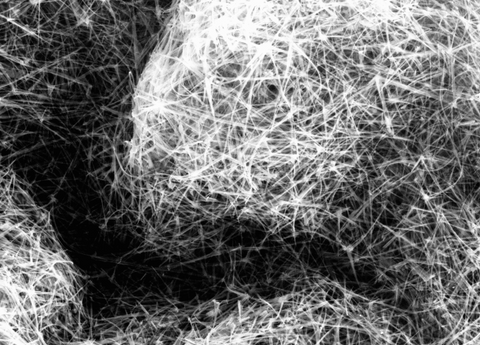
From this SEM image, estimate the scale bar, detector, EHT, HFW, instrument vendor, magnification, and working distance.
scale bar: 2000 nm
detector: InLens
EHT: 15 kV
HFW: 14.26 µm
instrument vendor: Zeiss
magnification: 20.83 K X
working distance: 7.8 mm